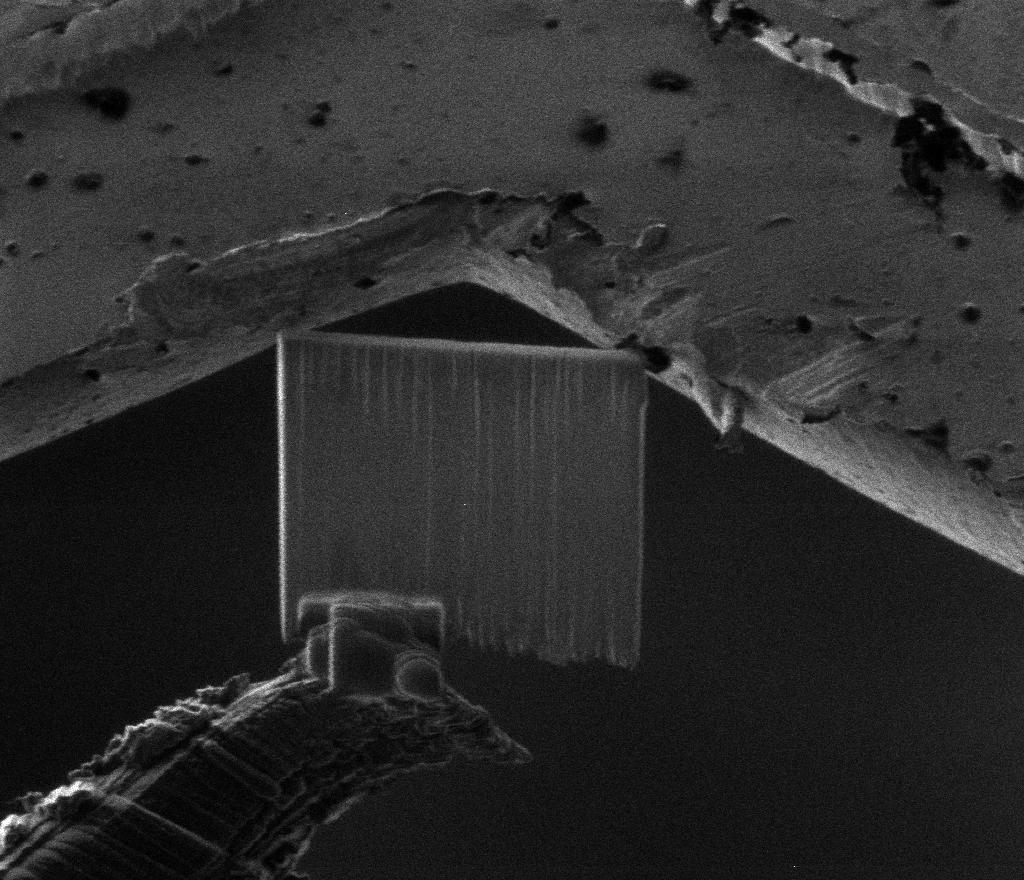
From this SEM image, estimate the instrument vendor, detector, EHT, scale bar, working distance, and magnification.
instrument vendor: FEI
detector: CDEM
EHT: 30 kV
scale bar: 10000 nm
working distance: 18.9 mm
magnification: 3 K X